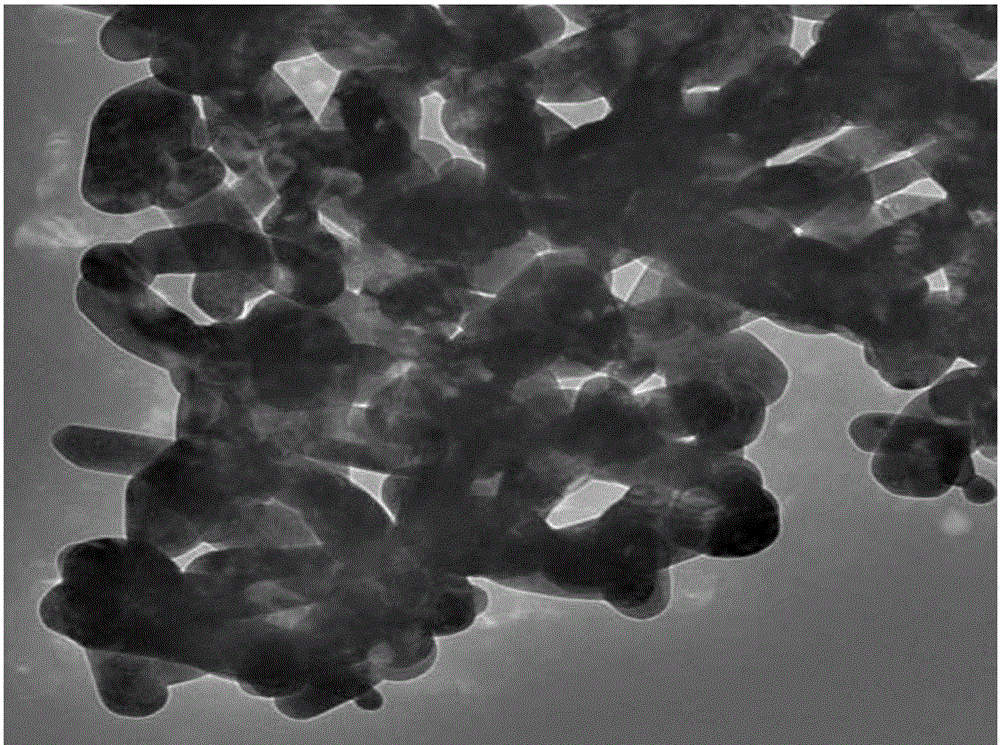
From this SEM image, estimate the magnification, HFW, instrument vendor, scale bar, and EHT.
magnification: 15 K X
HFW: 1.5 µm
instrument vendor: JEOL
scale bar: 200 nm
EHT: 200 kV